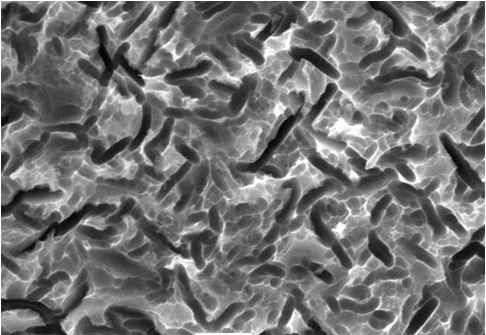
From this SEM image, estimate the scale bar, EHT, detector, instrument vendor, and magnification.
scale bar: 5000 nm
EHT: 20 kV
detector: SEI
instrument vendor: JEOL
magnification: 3 K X